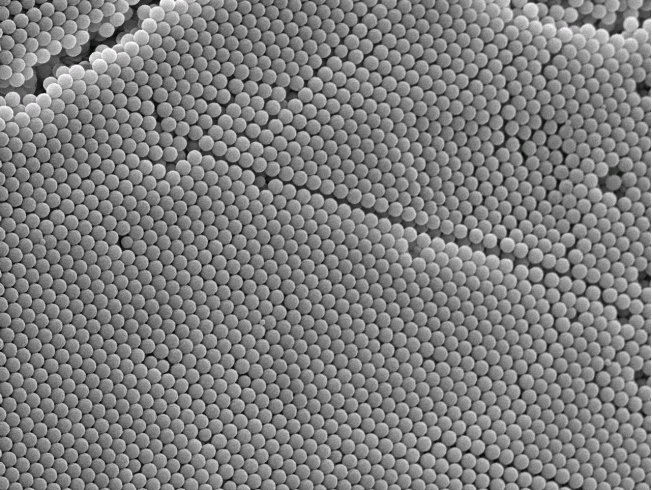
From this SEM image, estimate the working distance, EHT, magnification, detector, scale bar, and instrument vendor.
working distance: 7.2 mm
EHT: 5 kV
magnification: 10 K X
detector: SEI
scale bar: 1000 nm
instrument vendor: JEOL